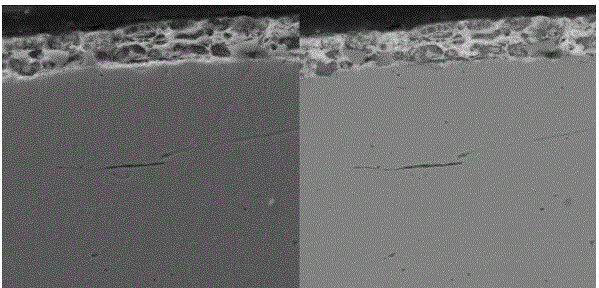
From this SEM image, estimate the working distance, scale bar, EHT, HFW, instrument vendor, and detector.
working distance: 13.7 mm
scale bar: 50000 nm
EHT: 30 kV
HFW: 104 µm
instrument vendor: Tescan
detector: SE, BSE